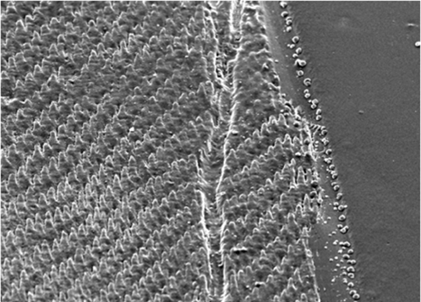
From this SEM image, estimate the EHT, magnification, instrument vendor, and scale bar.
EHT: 10 kV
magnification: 0.5 K X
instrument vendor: JEOL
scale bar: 20000 nm